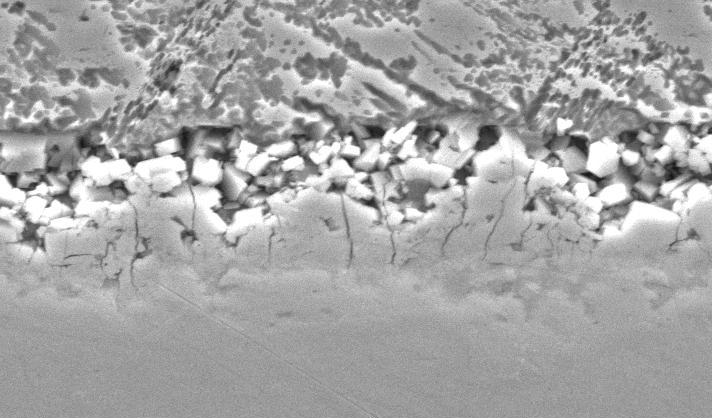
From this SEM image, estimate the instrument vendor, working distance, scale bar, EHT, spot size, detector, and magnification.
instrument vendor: FEI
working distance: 9.2 mm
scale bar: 10000 nm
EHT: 25 kV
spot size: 4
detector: SE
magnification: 5 K X